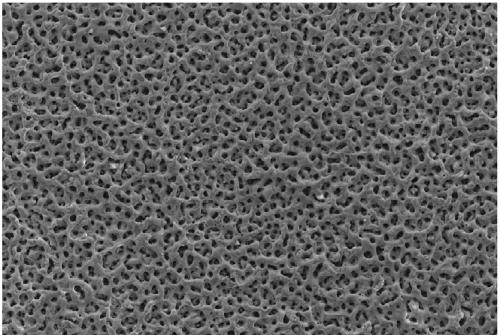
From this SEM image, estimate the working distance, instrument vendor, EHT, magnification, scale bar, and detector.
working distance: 12.1 mm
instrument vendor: Zeiss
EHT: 15 kV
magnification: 1 K X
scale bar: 10000 nm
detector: SE2